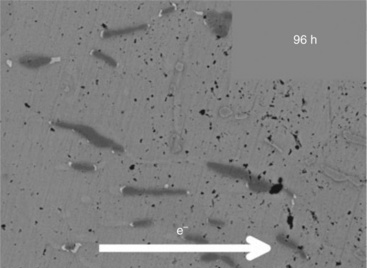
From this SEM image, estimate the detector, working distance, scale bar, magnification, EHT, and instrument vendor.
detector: COMPO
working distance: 15.2 mm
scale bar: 1000 nm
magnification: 5 K X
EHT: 10 kV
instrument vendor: JEOL